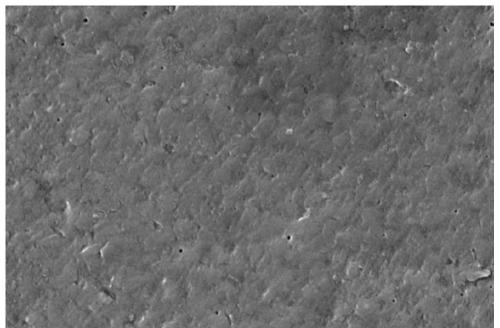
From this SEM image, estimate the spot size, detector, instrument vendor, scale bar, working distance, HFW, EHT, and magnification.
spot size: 3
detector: ETD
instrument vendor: FEI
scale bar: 2000 nm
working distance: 7.9 mm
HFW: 10.4 µm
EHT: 5 kV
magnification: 40 K X